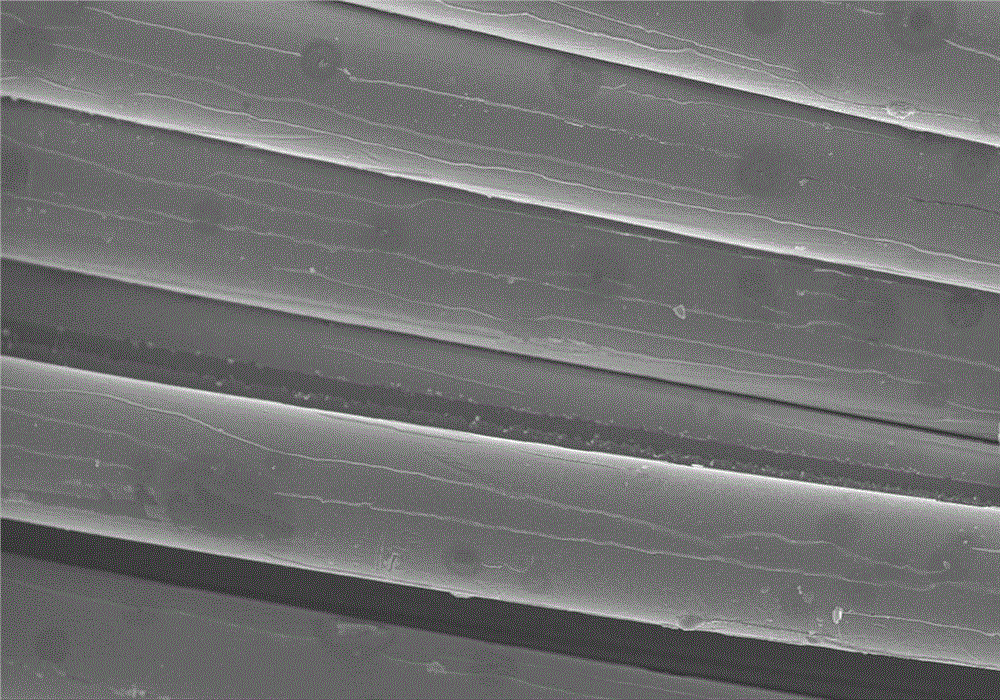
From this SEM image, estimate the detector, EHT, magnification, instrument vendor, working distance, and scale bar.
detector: SE(M)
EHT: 10 kV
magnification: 1 K X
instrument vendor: Hitachi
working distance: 7.5 mm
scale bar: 50000 nm